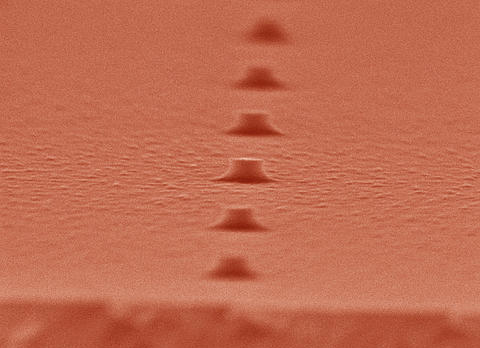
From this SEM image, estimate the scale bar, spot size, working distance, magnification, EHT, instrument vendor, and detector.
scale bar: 200 nm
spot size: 3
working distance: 5 mm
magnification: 80 K X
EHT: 20 kV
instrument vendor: FEI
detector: TLD